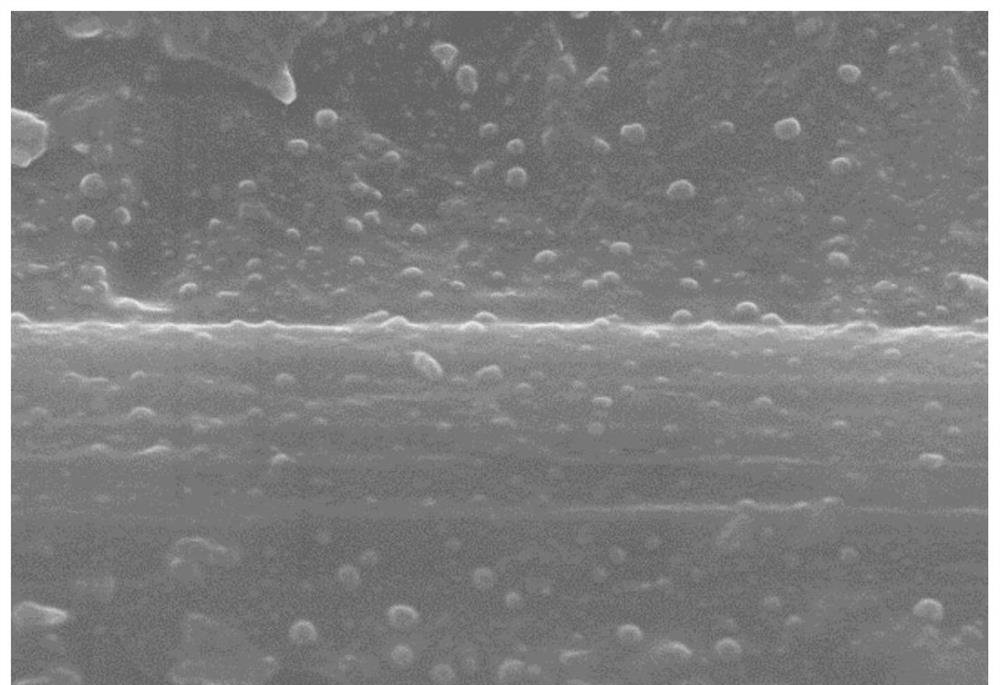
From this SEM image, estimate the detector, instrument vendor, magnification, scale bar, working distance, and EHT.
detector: SE(TUL)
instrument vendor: Hitachi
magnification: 20 K X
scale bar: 2000 nm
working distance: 8.4 mm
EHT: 10 kV